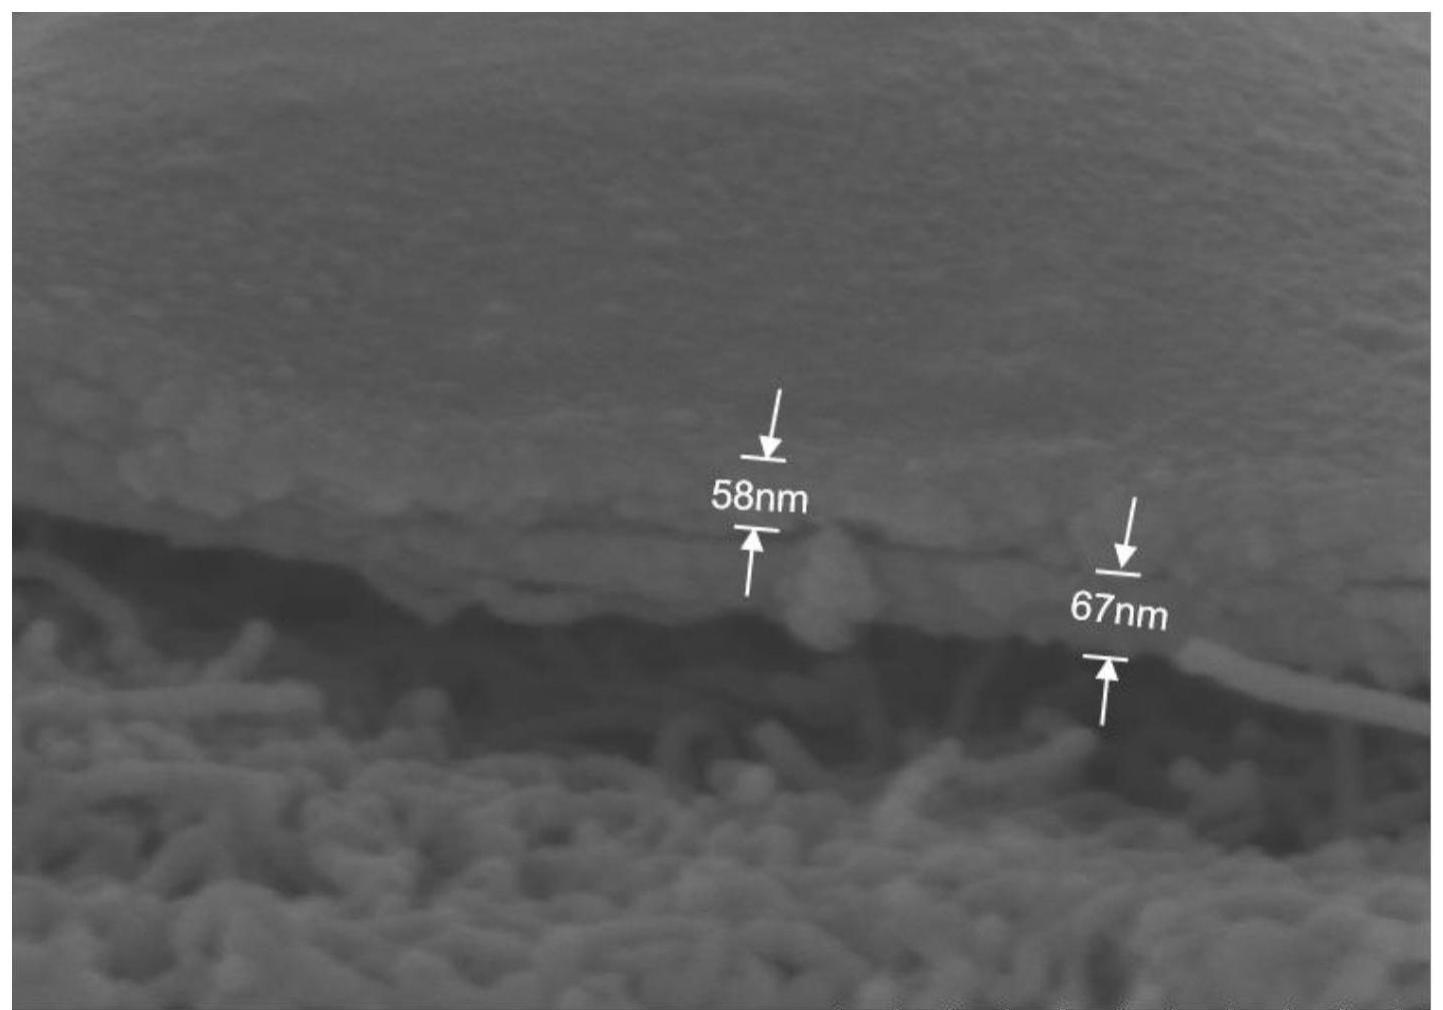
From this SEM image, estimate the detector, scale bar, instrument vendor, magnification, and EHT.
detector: SE(UL)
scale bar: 500 nm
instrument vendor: Hitachi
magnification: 100 K X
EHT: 4 kV